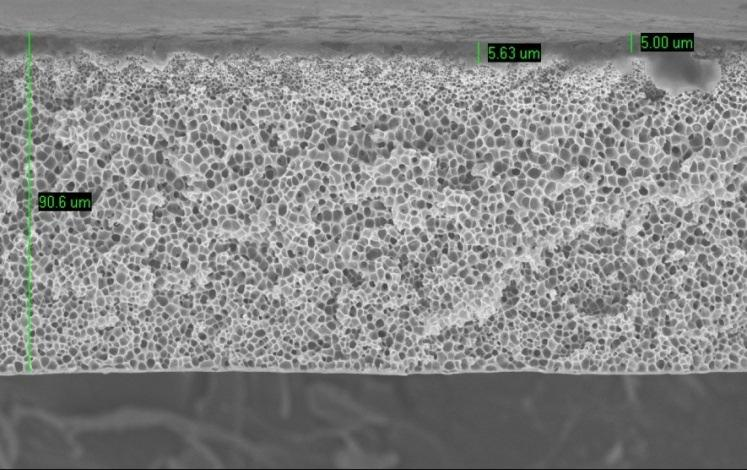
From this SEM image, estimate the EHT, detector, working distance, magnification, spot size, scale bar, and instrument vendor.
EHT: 12 kV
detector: SE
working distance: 15 mm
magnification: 0.5 K X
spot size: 3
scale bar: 20000 nm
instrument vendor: FEI